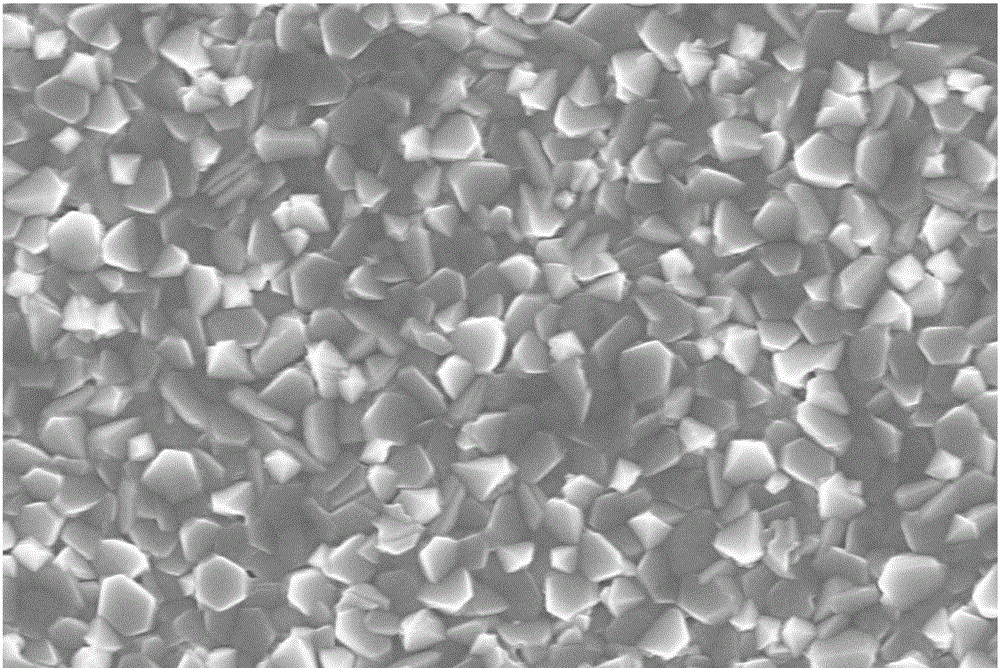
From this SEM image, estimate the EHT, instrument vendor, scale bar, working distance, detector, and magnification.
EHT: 8 kV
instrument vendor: Zeiss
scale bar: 200 nm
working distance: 8 mm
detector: InLens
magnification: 20 K X